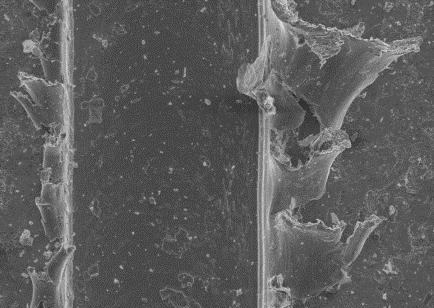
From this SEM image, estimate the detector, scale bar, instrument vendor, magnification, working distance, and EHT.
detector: SE1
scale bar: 100000 nm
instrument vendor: Zeiss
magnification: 0.25 K X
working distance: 29 mm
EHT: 20 kV